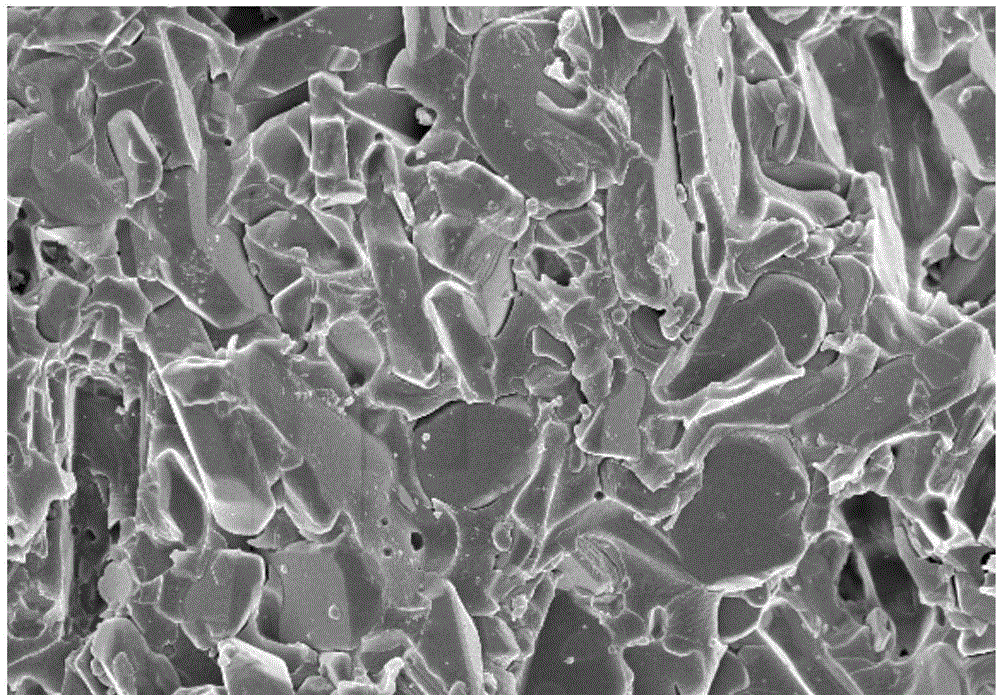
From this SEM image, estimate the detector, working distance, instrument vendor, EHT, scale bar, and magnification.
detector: SE(M)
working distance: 9.2 mm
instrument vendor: Hitachi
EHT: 5 kV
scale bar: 10000 nm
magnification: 5 K X